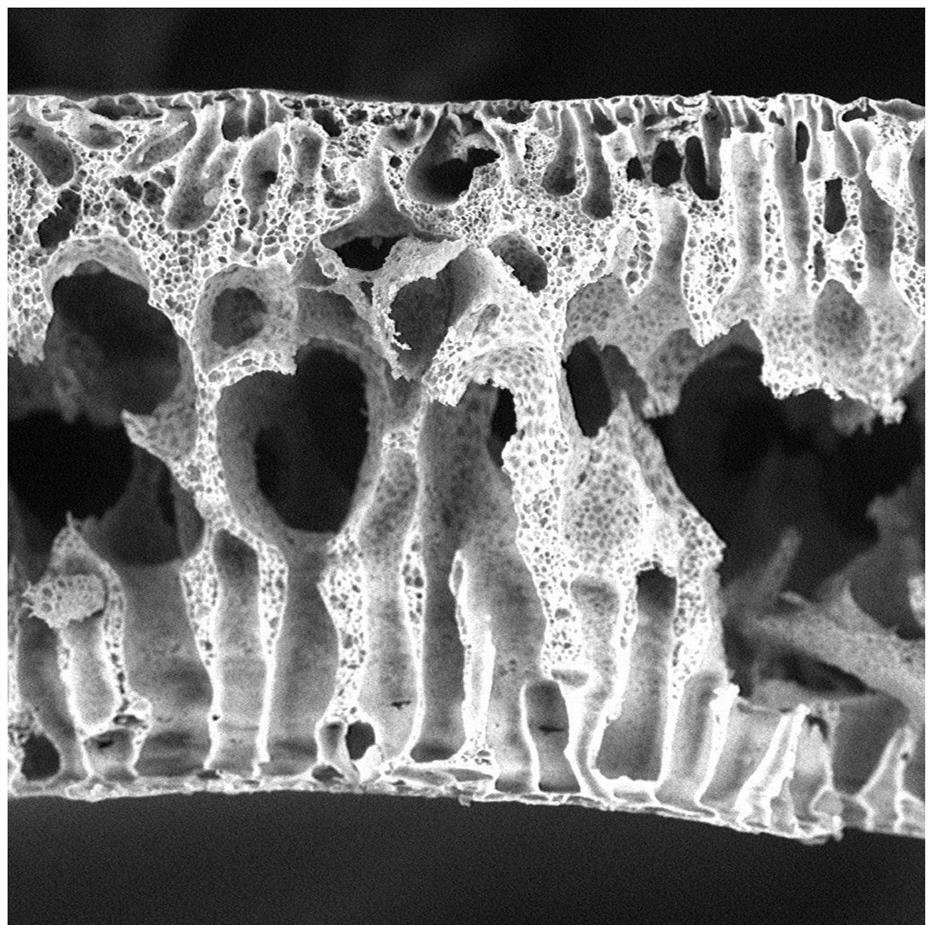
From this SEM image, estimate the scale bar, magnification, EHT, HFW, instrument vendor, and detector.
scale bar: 80000 nm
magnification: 1 K X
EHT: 15 kV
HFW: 268 µm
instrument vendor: Thermo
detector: BSD Full